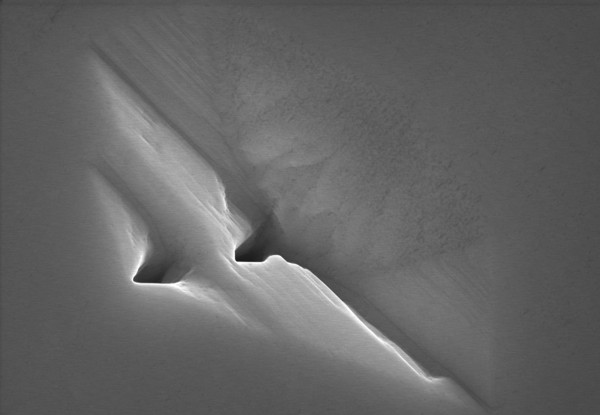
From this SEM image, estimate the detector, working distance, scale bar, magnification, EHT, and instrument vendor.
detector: SE(U)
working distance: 7.9 mm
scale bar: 2000 nm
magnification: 20 K X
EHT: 15 kV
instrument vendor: Hitachi